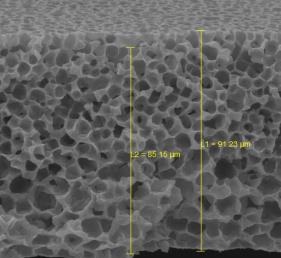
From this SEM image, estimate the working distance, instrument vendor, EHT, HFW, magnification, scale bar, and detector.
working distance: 30.78 mm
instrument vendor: Tescan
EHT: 30 kV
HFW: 115 µm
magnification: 1.2 K X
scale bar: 20000 nm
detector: SE